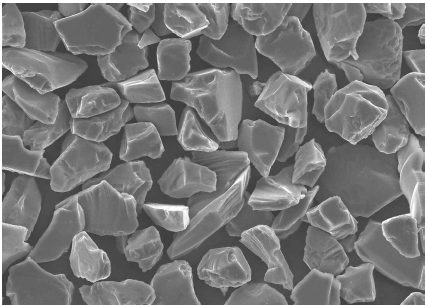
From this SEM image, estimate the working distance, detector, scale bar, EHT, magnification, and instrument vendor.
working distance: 19.3 mm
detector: SEI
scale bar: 10000 nm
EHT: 15 kV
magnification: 1 K X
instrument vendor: JEOL